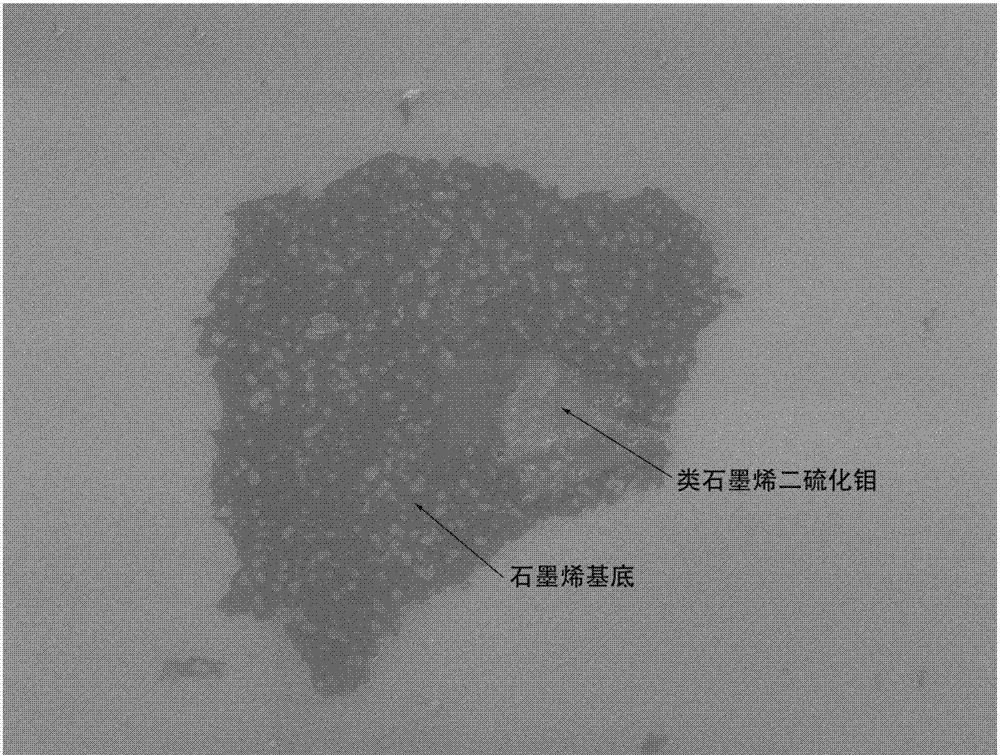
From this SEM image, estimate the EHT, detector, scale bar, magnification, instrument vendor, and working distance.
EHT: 5 kV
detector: SEI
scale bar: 1000 nm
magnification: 20 K X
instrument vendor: JEOL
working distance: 3.2 mm